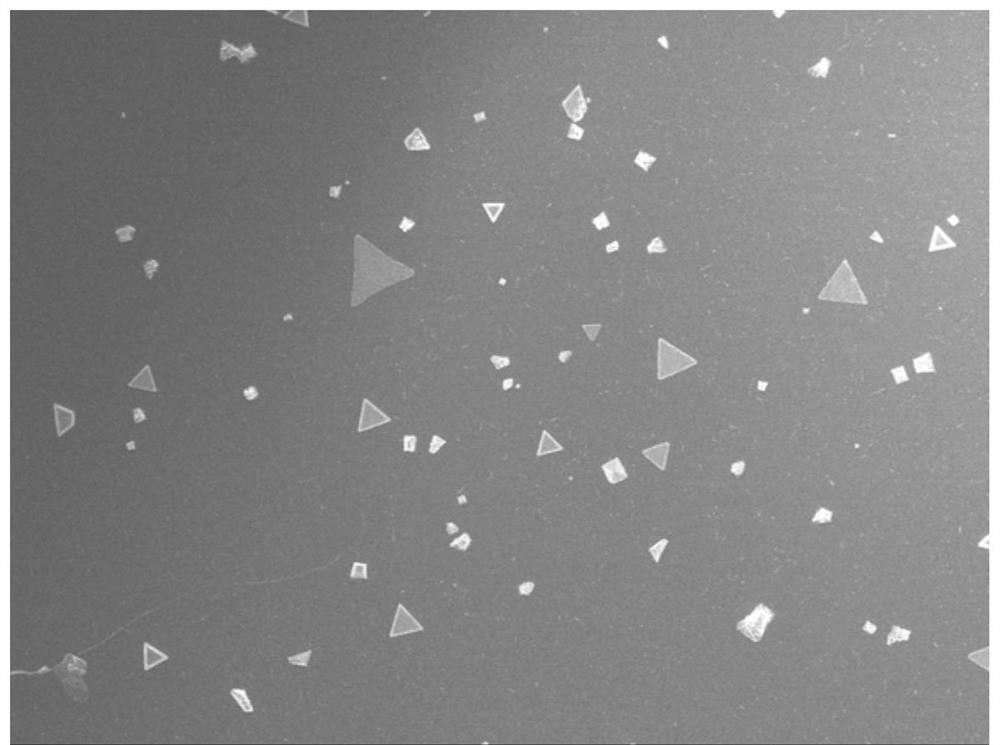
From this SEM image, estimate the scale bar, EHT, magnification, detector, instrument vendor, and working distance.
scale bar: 10000 nm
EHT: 10 kV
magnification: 0.5 K X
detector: SEI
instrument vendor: JEOL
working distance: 6.3 mm